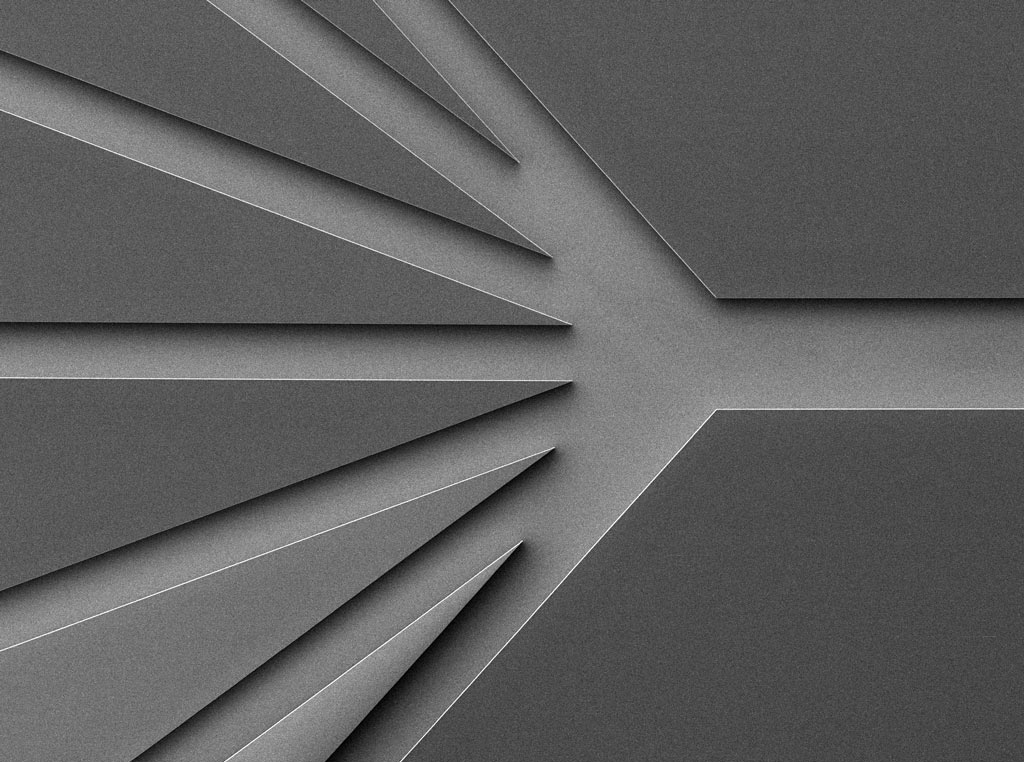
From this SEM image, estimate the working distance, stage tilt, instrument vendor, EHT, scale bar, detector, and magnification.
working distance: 20 mm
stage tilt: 0°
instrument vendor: JEOL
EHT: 2 kV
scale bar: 100000 nm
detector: SEI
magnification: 0.043 K X